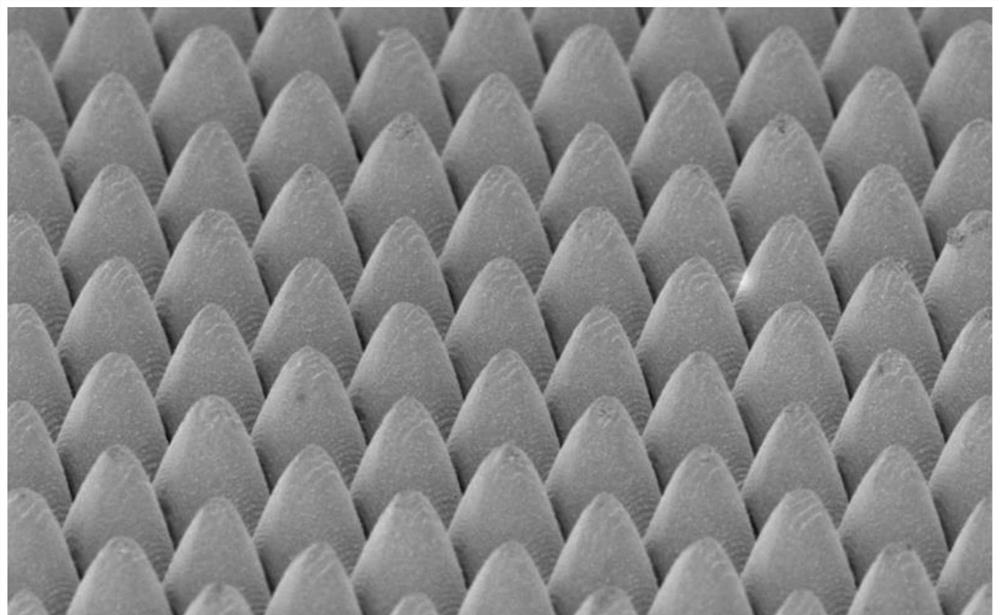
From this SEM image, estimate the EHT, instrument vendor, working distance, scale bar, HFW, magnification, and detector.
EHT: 10 kV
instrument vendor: other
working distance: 15.914 mm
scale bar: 80000 nm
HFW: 259 µm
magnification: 2 K X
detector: Mix 80%